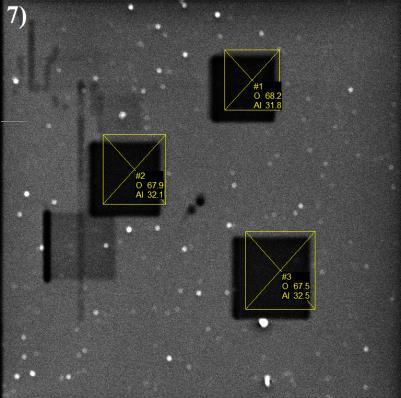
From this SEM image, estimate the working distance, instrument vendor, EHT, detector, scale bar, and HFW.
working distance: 15.79 mm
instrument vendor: Tescan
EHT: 5 kV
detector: SE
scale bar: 50000 nm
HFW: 196 µm